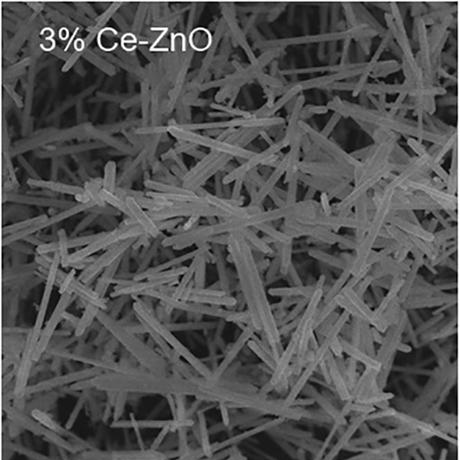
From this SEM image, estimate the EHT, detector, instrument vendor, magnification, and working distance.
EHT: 10 kV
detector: SE(M)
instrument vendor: Hitachi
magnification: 10 K X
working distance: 7.6 mm